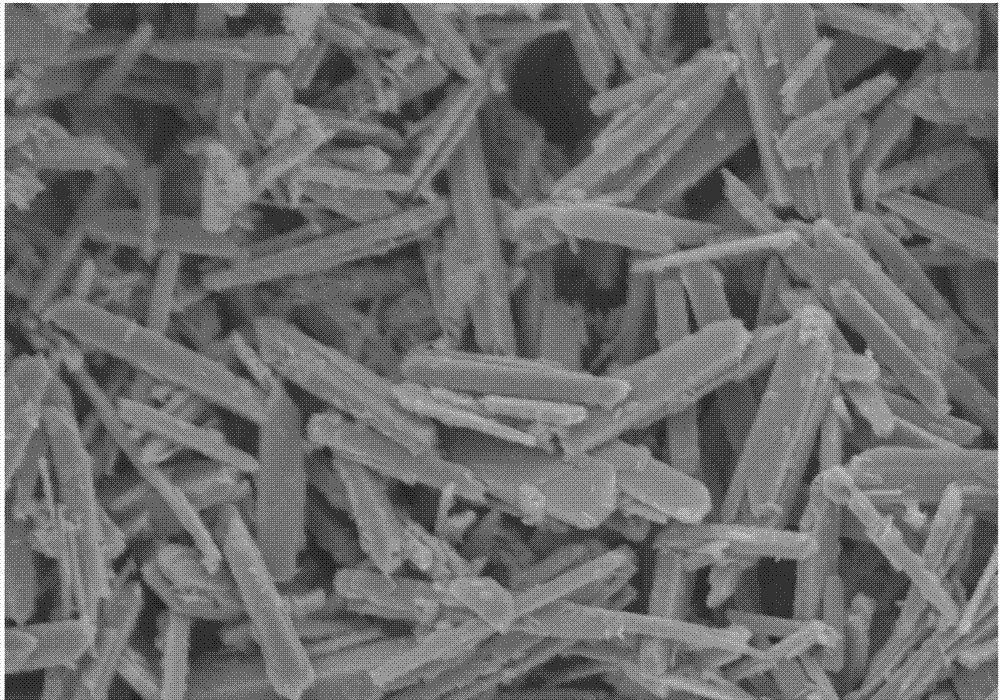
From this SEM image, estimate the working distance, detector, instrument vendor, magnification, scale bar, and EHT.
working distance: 6.8 mm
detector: SE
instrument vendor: Hitachi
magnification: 5 K X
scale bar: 10000 nm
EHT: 10 kV